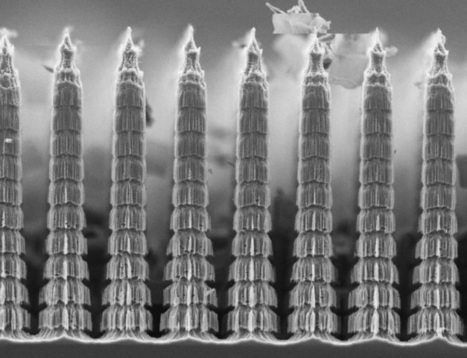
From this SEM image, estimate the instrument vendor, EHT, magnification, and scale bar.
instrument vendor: other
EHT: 10 kV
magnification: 0.8 K X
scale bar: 20000 nm